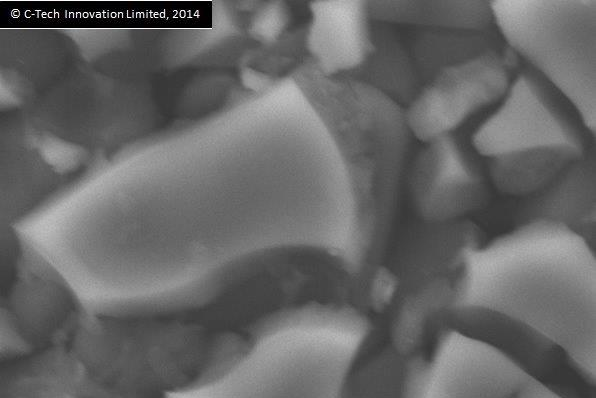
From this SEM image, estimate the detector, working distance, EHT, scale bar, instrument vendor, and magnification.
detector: SEI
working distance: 10 mm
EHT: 20 kV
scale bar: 2000 nm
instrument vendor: JEOL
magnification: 6 K X